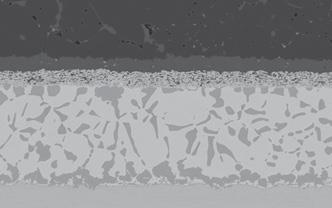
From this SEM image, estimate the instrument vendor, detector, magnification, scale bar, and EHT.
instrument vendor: Zeiss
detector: QBSD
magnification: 1.3 K X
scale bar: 30000 nm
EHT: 20 kV